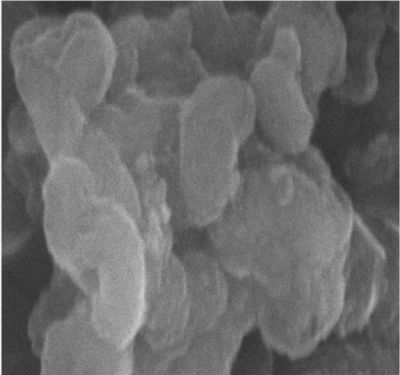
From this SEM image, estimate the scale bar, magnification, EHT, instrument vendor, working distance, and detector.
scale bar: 1000 nm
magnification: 50 K X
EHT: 20 kV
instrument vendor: Hitachi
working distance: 5.7 mm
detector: SE